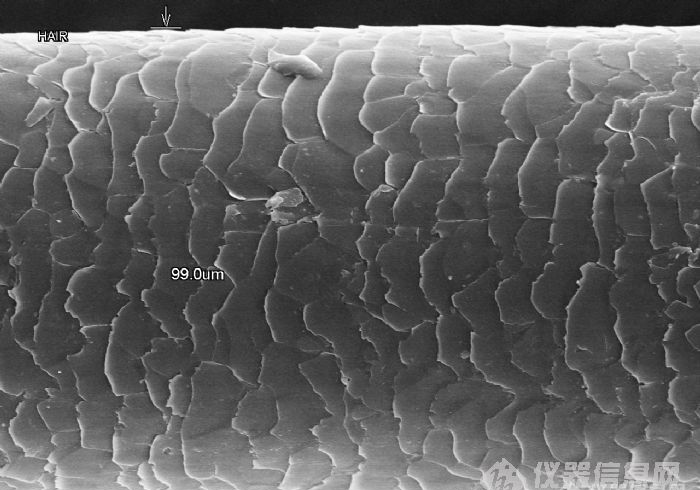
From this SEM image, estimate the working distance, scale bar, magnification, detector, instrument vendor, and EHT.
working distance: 8.3 mm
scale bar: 50000 nm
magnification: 0.9 K X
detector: SE(M)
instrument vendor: Hitachi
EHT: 15 kV